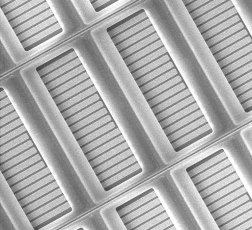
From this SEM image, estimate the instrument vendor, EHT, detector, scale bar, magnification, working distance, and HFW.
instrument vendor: Tescan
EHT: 15 kV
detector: SE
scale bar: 50000 nm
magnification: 0.662 K X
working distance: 14.84 mm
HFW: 313 µm